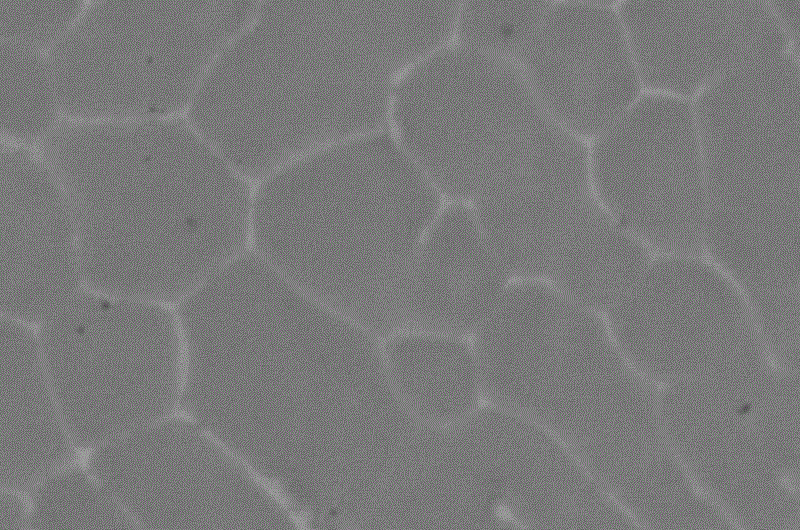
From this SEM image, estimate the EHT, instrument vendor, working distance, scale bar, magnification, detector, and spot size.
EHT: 10 kV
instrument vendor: FEI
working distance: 7.9 mm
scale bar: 3000 nm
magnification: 35 K X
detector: CBS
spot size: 3.5